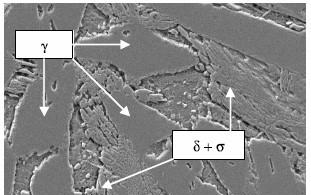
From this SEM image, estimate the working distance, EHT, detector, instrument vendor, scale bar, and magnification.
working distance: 12 mm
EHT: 20 kV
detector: SE1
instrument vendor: Zeiss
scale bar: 2000 nm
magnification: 2.5 K X